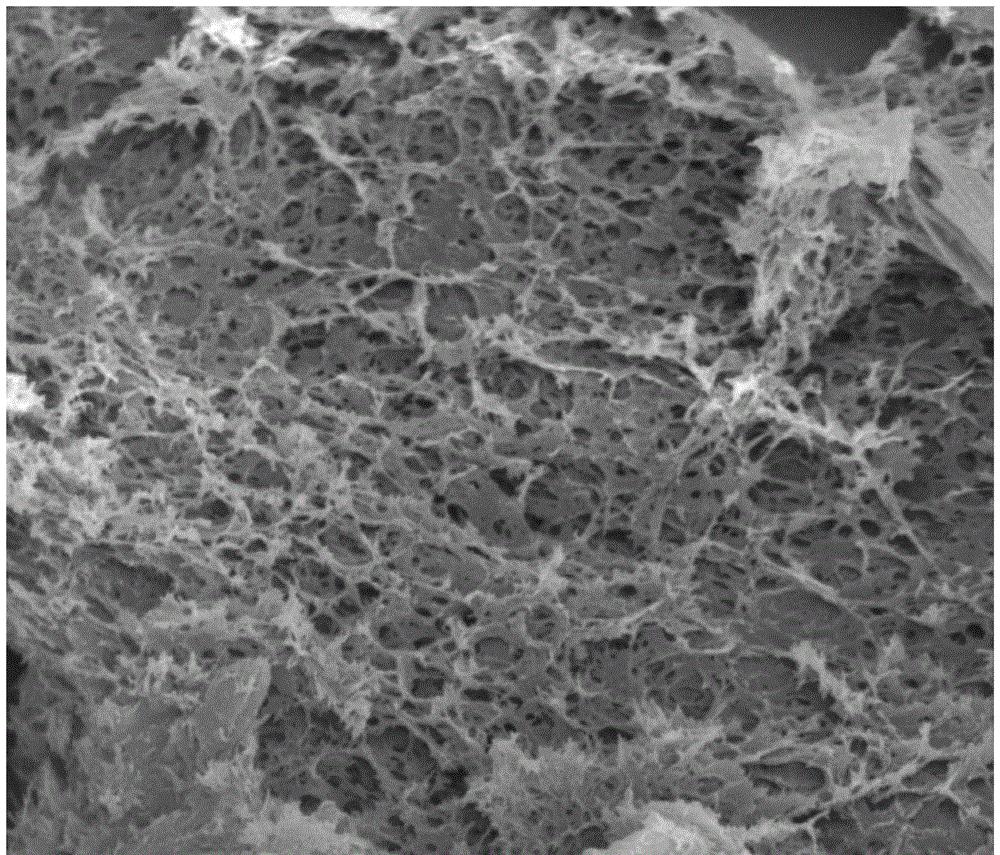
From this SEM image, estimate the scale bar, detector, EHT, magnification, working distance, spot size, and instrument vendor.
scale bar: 5000 nm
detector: ETD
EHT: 12.5 kV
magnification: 5 K X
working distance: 9.7 mm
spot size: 4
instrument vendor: FEI